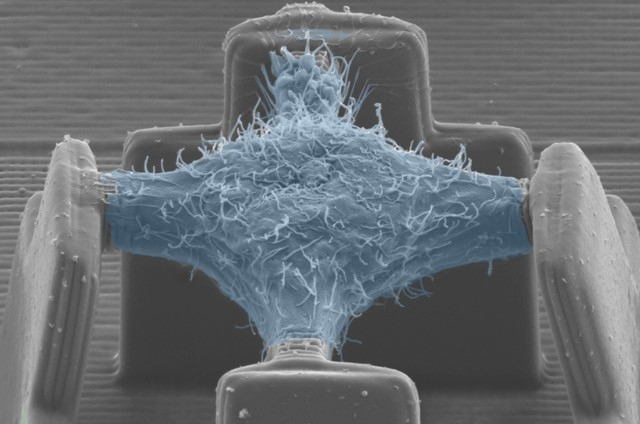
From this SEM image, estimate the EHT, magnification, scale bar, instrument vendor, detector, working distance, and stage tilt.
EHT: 5 kV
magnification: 3.74 K X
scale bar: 2000 nm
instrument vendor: Zeiss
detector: SE2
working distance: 16.2 mm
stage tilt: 45°